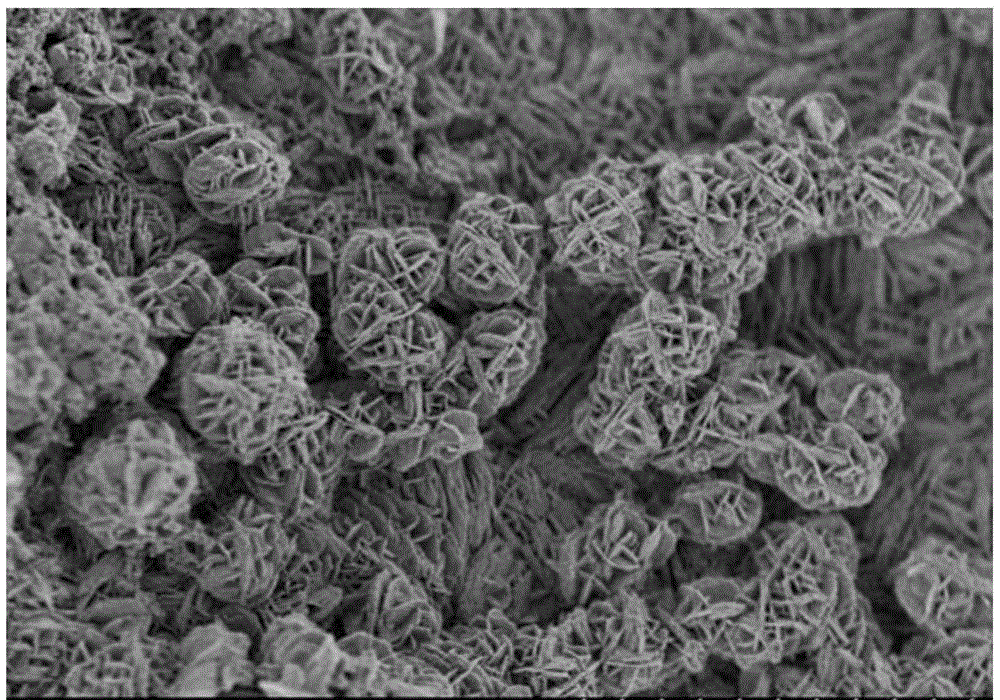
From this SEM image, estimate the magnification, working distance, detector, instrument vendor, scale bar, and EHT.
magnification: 10 K X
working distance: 1.8 mm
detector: SE(U,LA100)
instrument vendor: Hitachi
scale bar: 5000 nm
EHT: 1 kV